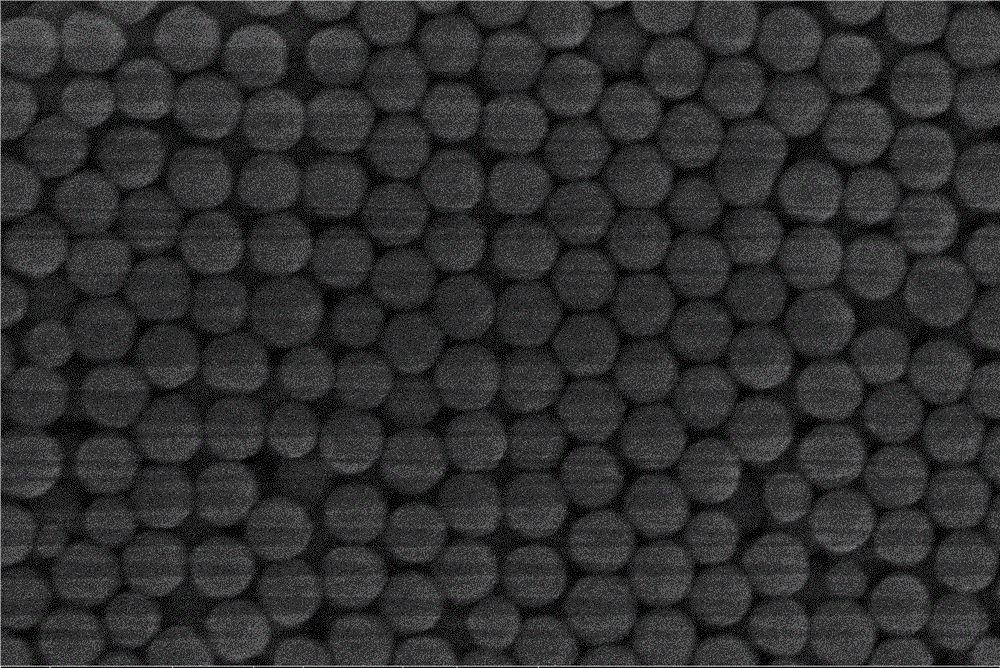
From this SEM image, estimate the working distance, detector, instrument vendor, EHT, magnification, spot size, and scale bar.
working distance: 4.6 mm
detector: TLD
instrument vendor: FEI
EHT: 5 kV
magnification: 100 K X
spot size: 3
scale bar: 1000 nm